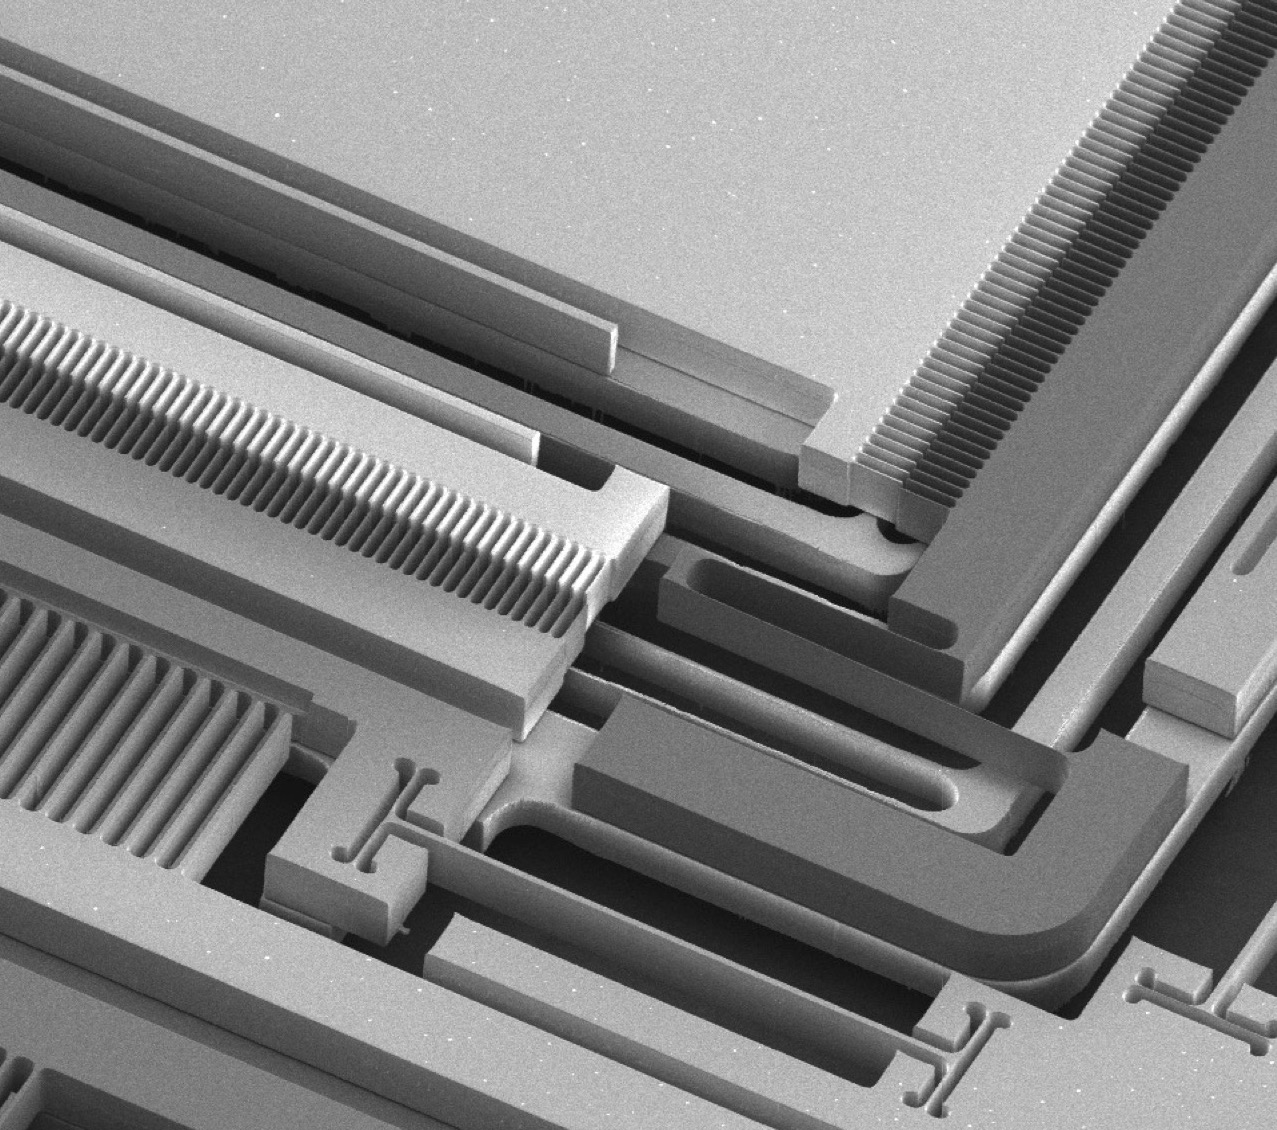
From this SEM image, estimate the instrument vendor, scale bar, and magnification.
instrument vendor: JEOL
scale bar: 100000 nm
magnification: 0.1 K X